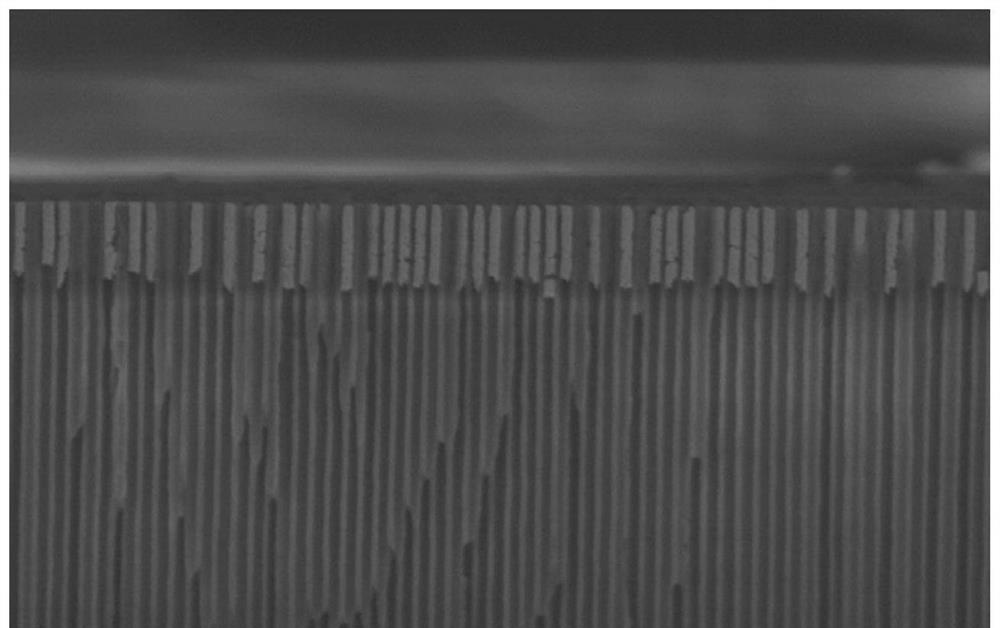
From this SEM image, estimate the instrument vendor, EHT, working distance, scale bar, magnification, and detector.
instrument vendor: Hitachi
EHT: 3 kV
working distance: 2.4 mm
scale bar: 2000 nm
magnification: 20 K X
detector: LA30(U)